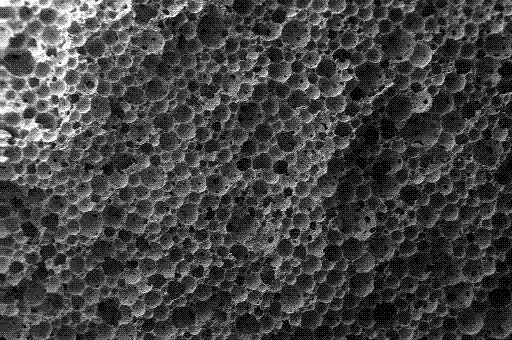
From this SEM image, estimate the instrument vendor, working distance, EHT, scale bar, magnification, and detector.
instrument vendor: Zeiss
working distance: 10 mm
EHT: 20 kV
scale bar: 200000 nm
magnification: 0.025 K X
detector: SE1